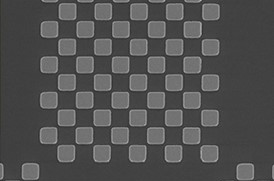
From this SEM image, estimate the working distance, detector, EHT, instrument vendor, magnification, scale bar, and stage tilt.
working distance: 4.3 mm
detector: TLD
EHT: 5 kV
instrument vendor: FEI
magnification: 8 K X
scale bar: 5000 nm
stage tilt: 0°